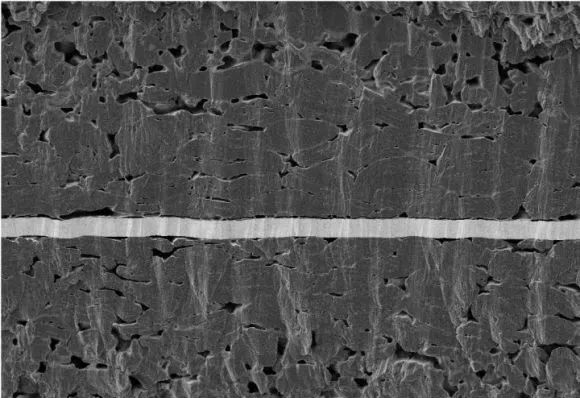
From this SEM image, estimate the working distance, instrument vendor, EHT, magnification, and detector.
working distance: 4.1 mm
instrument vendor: Zeiss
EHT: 3 kV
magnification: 0.601 K X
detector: SE2